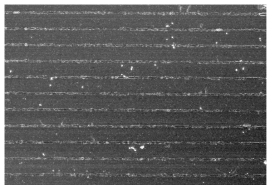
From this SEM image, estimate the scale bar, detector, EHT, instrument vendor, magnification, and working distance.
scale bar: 50000 nm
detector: SE(U)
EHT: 10 kV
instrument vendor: Hitachi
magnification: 1 K X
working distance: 8.1 mm